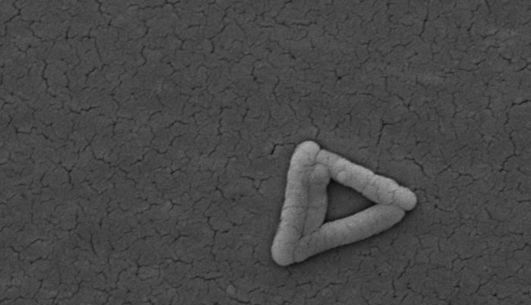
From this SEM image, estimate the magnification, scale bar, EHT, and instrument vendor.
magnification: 10 K X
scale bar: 2000 nm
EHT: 30 kV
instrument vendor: FEI